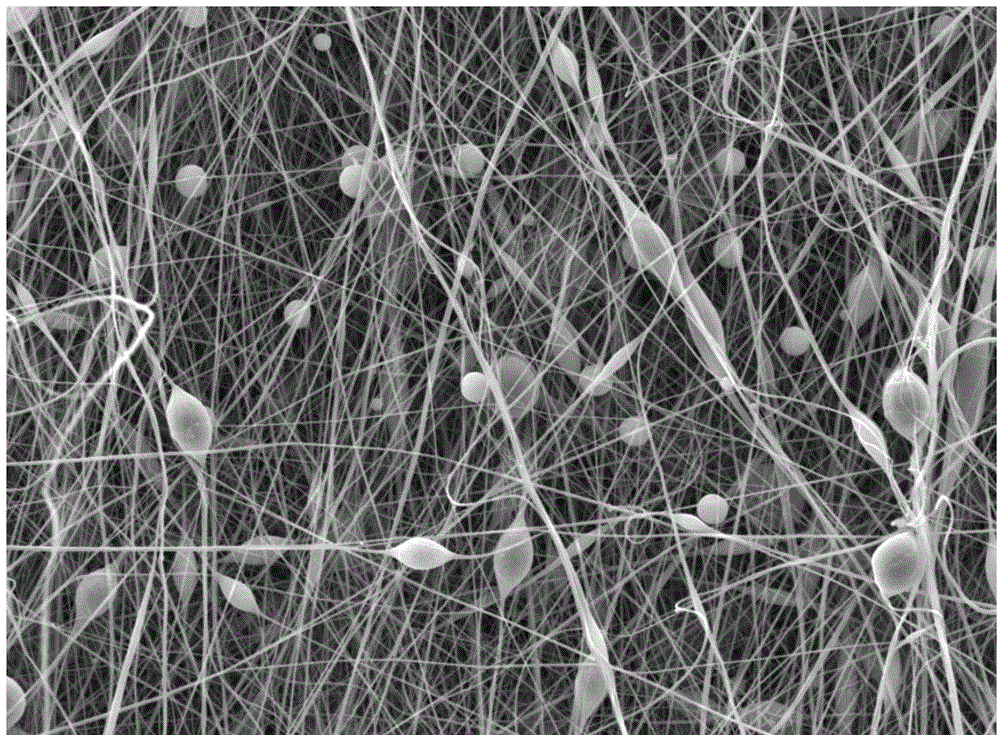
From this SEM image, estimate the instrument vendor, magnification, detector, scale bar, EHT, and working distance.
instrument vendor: JEOL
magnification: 2 K X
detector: LED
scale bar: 10000 nm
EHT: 10 kV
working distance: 9.7 mm